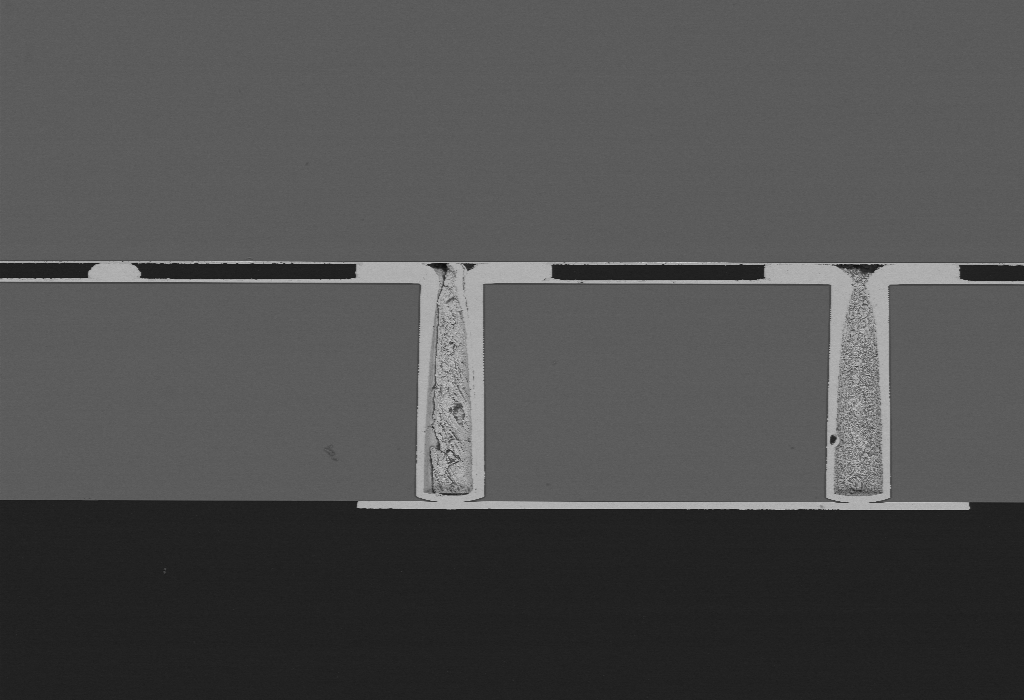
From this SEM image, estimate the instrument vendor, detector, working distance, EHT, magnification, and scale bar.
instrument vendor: Zeiss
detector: CZ BSD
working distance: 7.1 mm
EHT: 10 kV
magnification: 0.15 K X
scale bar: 100000 nm